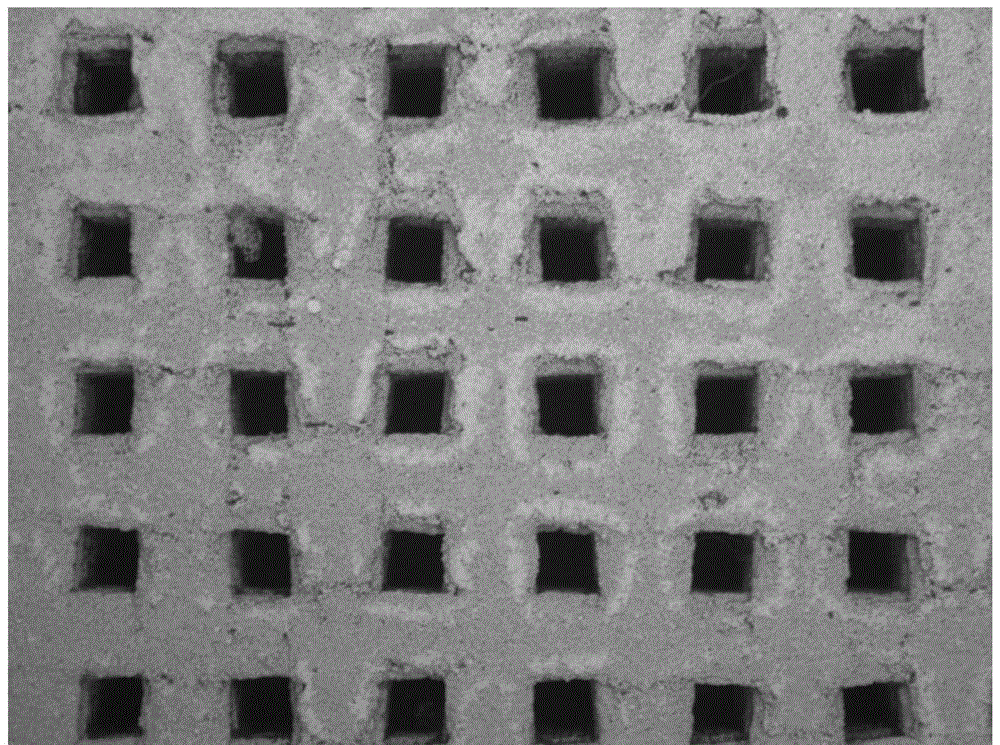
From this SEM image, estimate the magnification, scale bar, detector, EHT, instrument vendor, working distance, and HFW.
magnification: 0.04 K X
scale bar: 2000000 nm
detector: BSE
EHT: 25 kV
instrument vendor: Tescan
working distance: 15.5 mm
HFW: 6920 µm